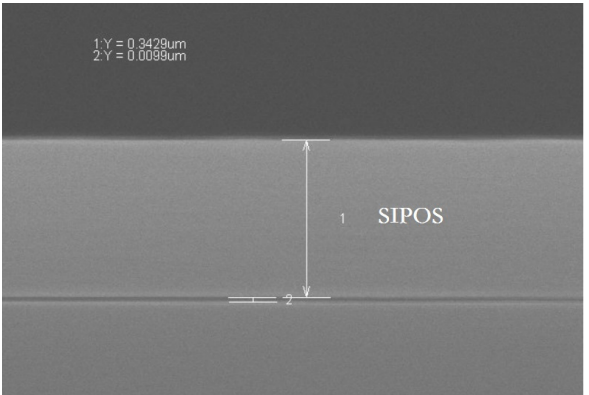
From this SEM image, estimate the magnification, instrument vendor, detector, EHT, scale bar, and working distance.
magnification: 100 K X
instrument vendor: Hitachi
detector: SE(UL)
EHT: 8 kV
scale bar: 500 nm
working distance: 9.4 mm